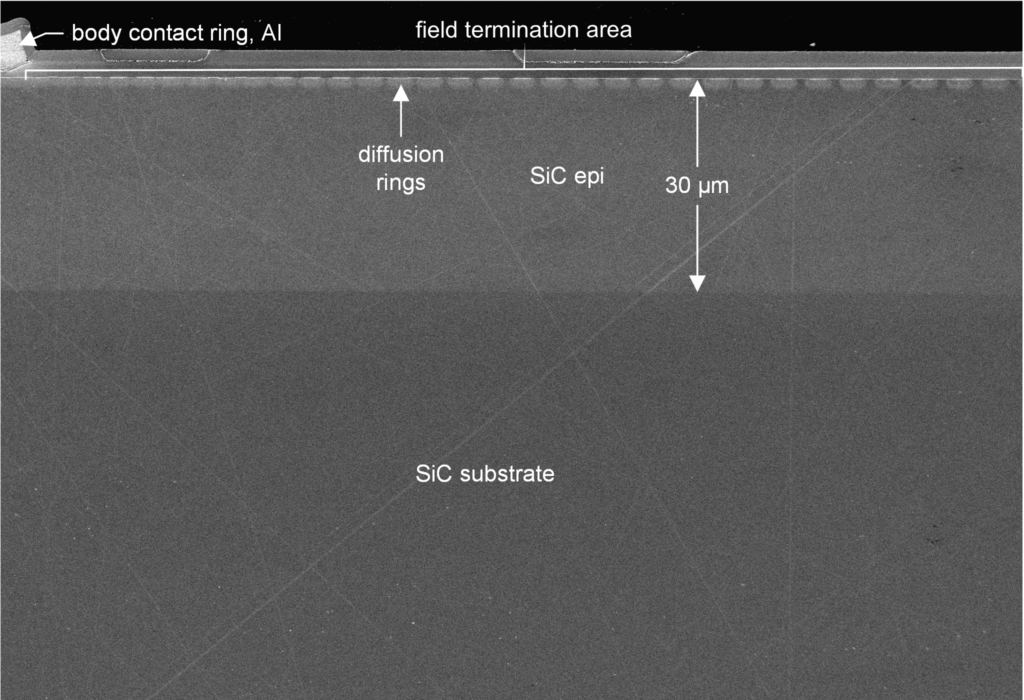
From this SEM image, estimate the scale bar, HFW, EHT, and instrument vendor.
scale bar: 20000 nm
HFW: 145.5 µm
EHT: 1 kV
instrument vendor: Zeiss